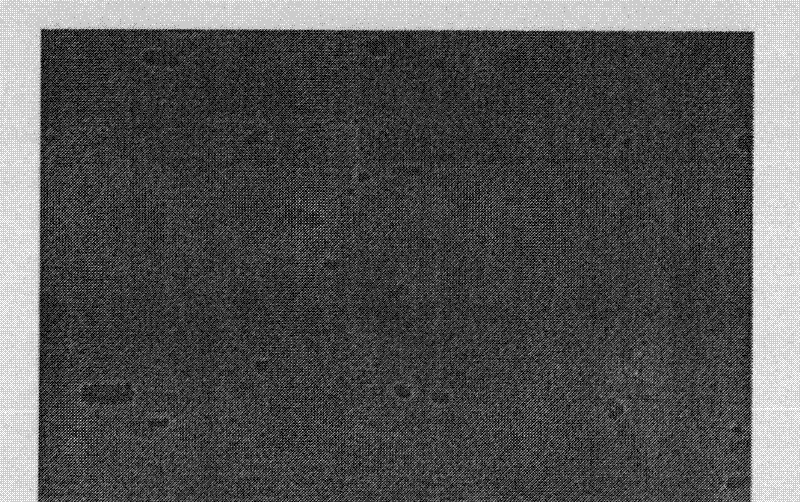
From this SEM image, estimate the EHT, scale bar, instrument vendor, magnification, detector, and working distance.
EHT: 10 kV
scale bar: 1000 nm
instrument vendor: Hitachi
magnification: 30 K X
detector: SE(M)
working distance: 13.5 mm